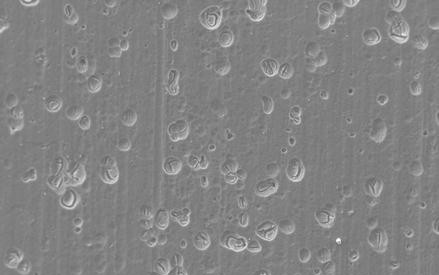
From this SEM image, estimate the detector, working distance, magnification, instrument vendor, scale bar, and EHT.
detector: SE1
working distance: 10.5 mm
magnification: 1 K X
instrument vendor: Zeiss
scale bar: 10000 nm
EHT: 15 kV